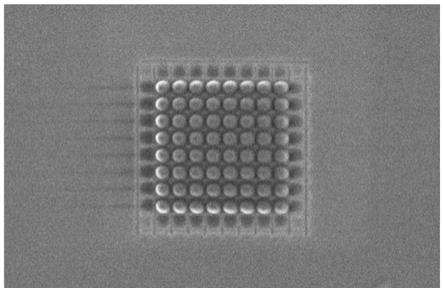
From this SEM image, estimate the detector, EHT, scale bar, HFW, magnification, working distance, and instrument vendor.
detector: TLD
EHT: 2 kV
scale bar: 1000 nm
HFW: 5.18 µm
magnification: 80 K X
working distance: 4 mm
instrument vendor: FEI